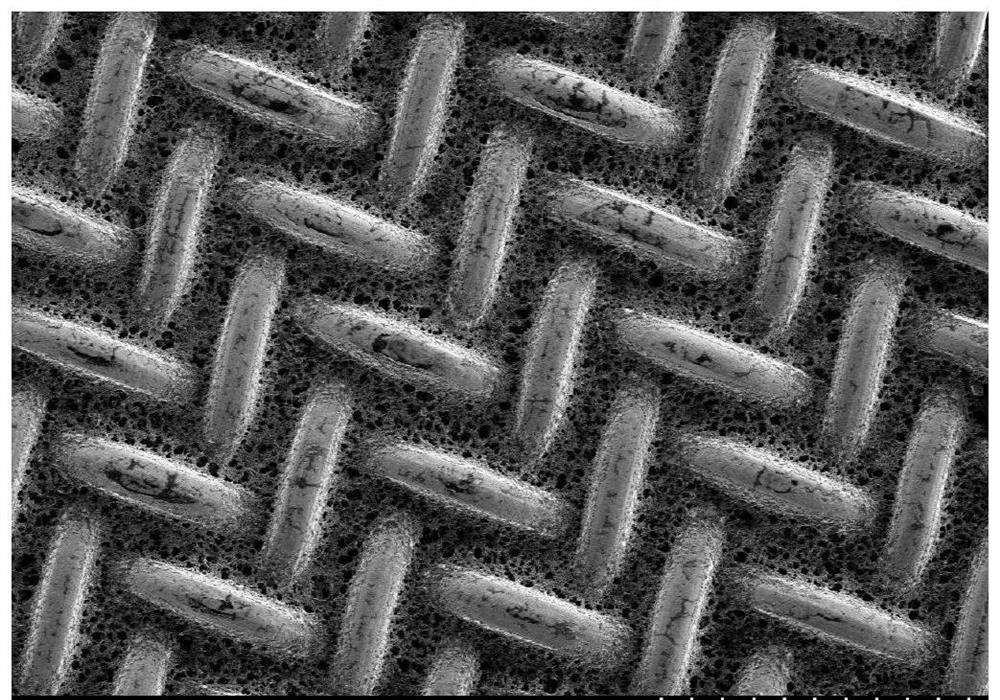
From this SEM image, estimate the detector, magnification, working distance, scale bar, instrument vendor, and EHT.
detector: SE(M)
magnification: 0.2 K X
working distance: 7.2 mm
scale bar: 200000 nm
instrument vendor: Hitachi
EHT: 3 kV